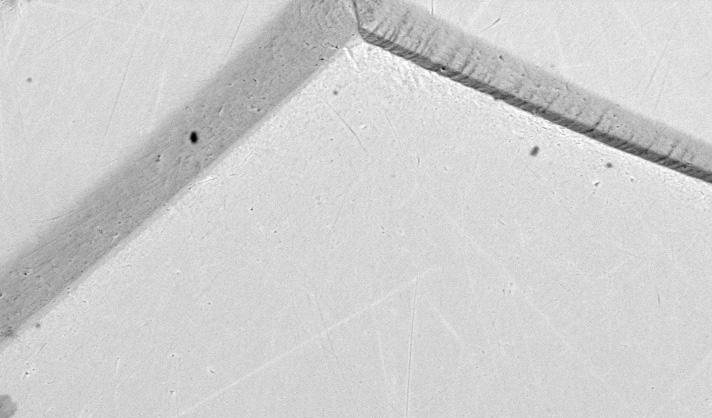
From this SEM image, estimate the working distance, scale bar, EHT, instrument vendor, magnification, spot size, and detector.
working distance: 11.4 mm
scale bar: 50000 nm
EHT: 20 kV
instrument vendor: FEI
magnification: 0.5 K X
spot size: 5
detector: BSE